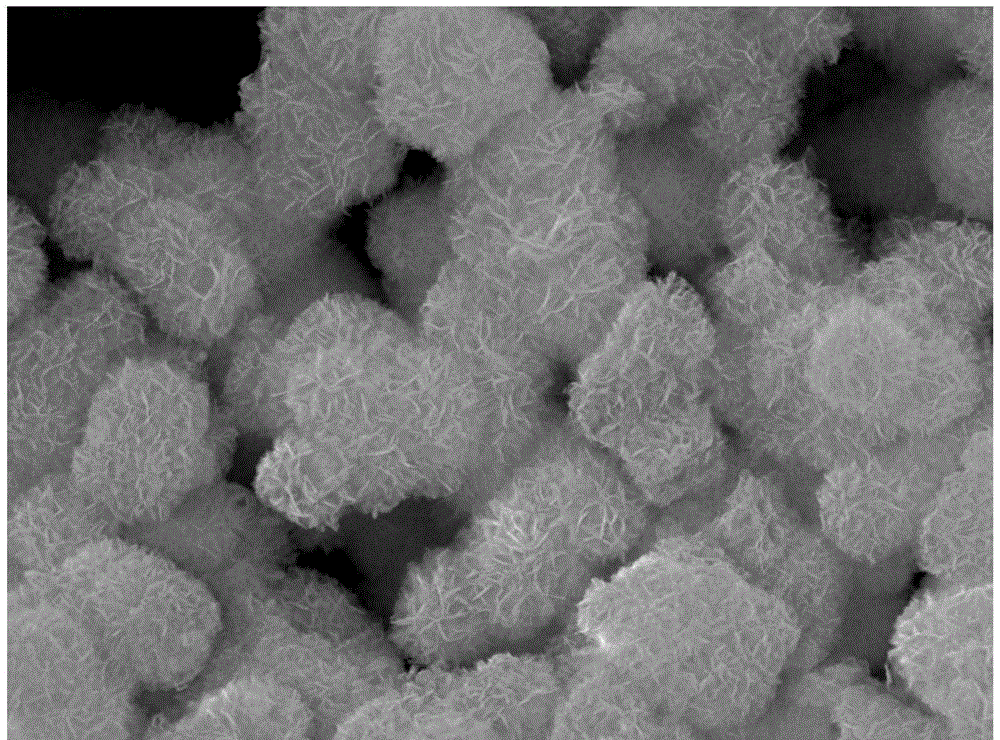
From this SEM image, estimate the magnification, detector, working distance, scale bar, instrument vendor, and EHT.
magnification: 20 K X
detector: SEI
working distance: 9.9 mm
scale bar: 1000 nm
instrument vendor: JEOL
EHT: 15 kV